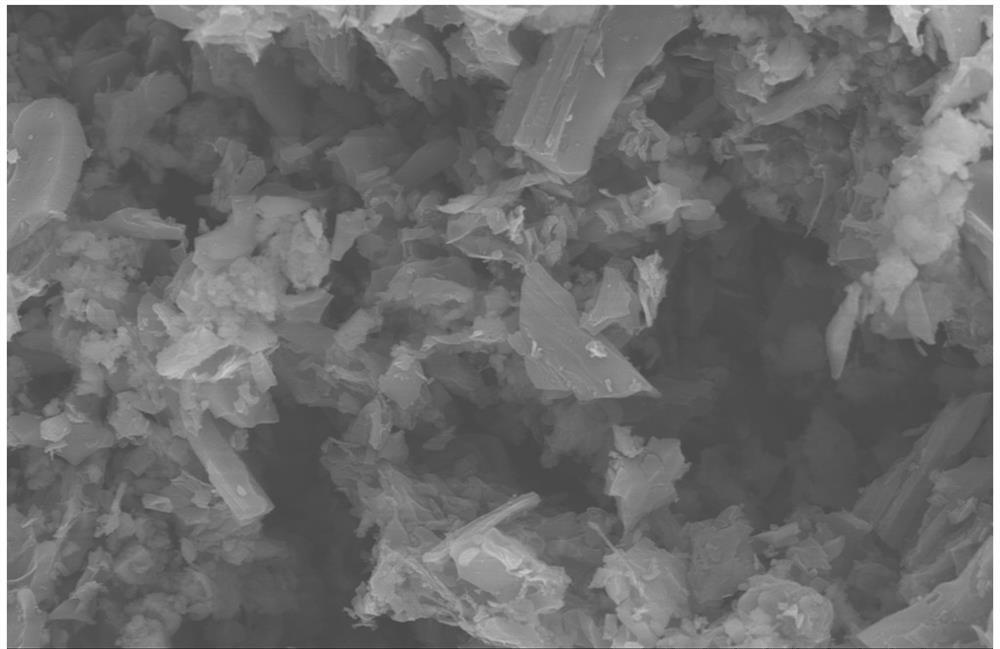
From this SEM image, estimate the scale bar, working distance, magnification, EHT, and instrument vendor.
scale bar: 20000 nm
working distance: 15.5 mm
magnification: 2 K X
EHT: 10 kV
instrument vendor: Hitachi